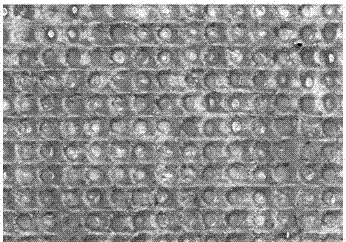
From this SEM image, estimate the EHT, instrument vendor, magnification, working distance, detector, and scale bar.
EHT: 20 kV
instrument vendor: Hitachi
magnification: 0.1 K X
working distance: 13.5 mm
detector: SE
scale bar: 500000 nm